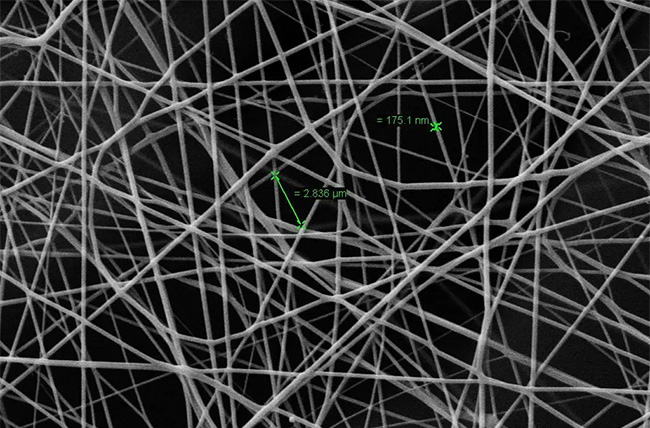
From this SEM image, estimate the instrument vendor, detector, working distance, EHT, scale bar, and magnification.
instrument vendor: Zeiss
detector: SE1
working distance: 5.5 mm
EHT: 10 kV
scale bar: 3000 nm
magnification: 3.43 K X